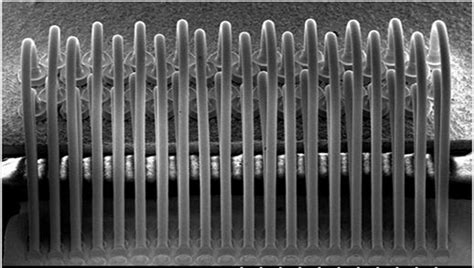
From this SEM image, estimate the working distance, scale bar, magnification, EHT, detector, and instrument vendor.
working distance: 12.7 mm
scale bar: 500000 nm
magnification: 0.1 K X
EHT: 20 kV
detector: SE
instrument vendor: Hitachi